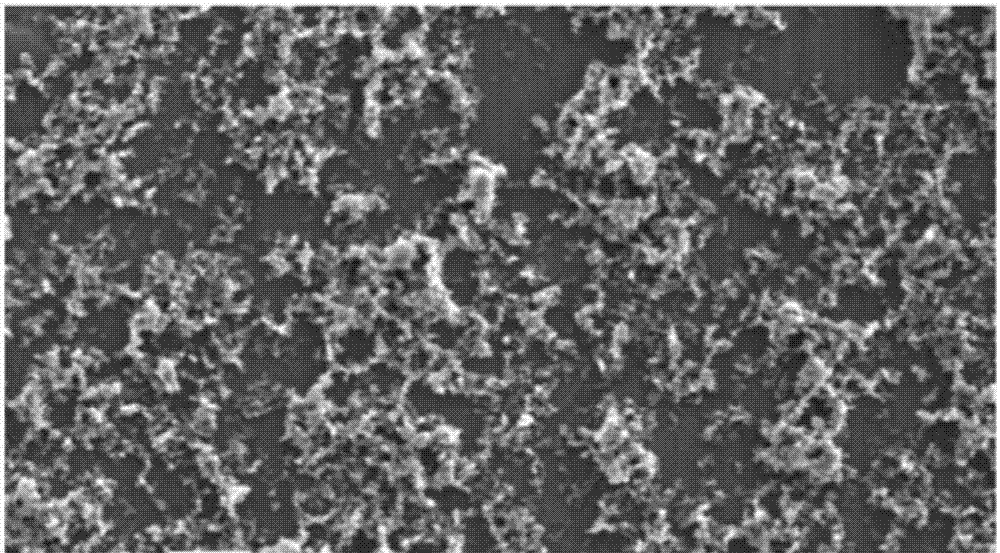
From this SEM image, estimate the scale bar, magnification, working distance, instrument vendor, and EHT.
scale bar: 3330 nm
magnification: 3 K X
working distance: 9.4 mm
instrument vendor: Hitachi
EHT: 5 kV